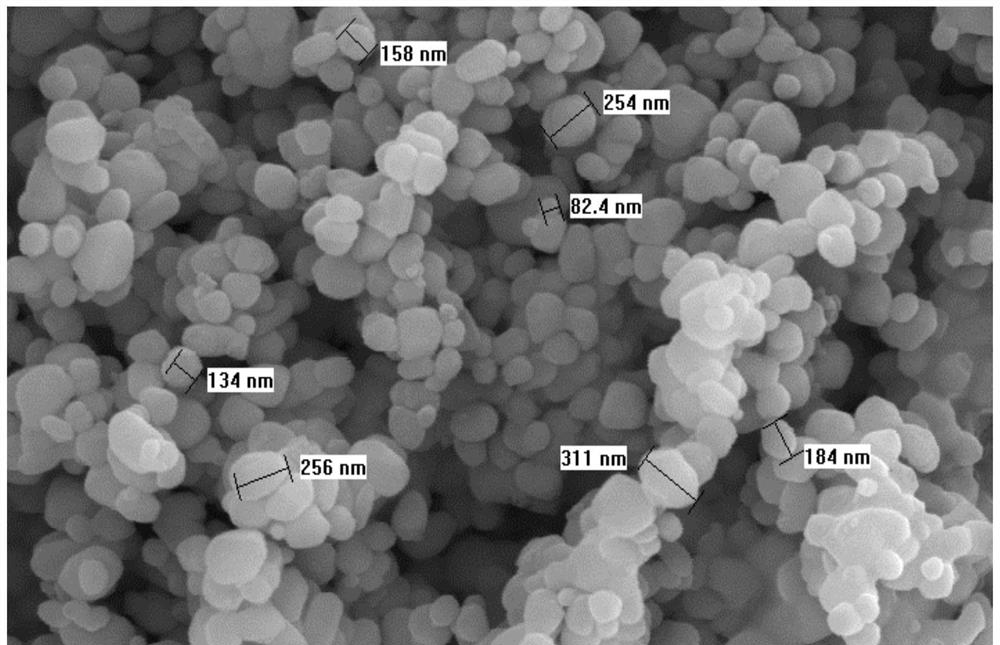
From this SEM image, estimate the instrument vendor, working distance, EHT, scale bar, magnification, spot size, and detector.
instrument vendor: FEI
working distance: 7.5 mm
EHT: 15 kV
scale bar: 1000 nm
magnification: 20 K X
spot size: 3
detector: SE